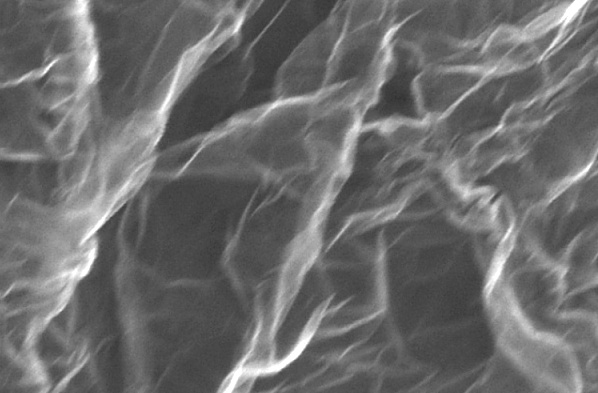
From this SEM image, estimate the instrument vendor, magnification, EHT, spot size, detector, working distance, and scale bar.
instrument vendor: FEI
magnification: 160 K X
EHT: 5 kV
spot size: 4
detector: TLD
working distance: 5.3 mm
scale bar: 500 nm